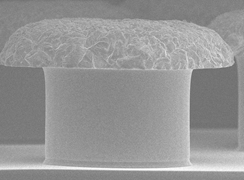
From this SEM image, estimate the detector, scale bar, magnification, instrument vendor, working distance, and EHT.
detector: SEI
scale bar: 10000 nm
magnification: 1 K X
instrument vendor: JEOL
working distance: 8 mm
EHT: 10 kV